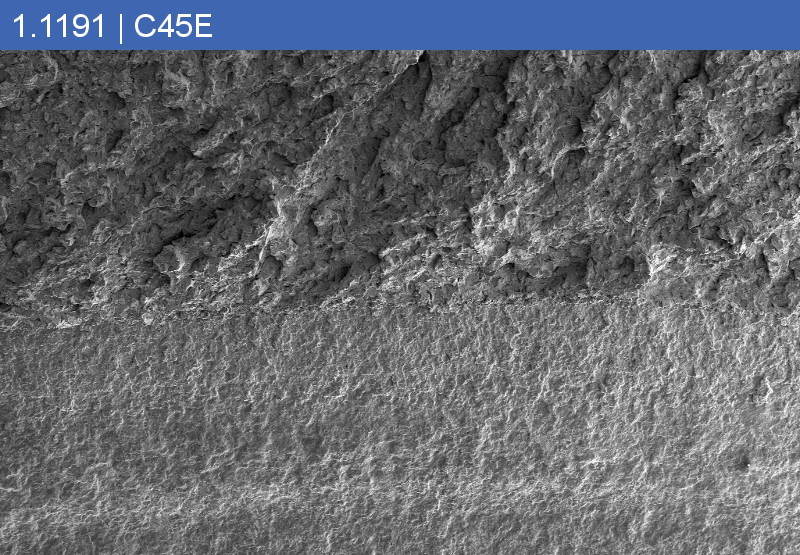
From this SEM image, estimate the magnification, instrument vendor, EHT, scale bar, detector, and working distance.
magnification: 0.02 K X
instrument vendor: Zeiss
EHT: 15 kV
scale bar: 200000 nm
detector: SE2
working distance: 13.1 mm